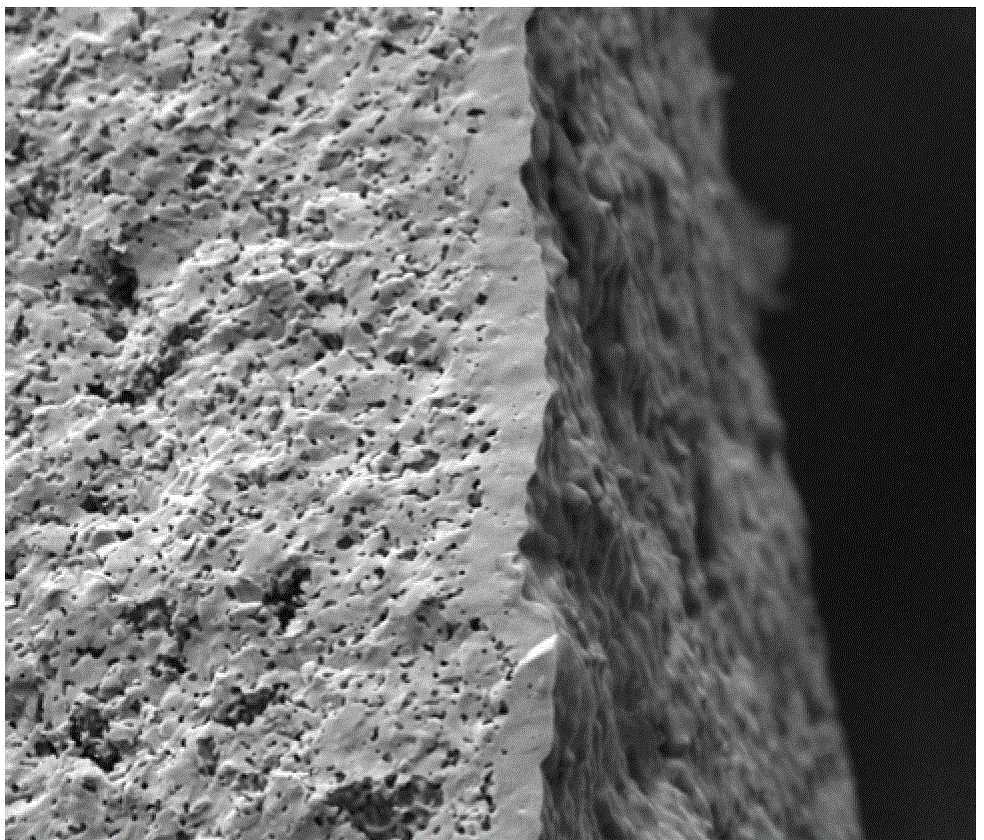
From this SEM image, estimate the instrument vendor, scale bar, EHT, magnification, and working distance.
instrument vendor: FEI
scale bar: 100000 nm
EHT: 20 kV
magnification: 0.6 K X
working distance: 12.3 mm